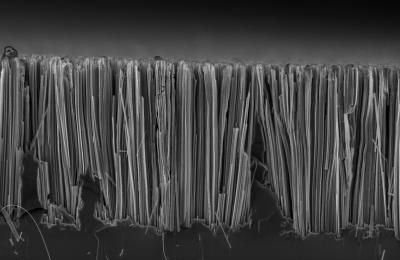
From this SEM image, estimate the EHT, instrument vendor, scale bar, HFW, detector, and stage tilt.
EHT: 3 kV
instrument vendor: FEI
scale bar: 10000 nm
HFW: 21.3 µm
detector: TLD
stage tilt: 0°